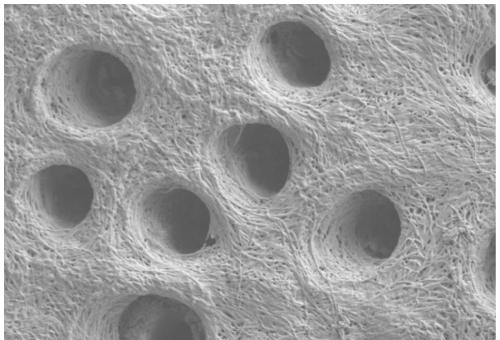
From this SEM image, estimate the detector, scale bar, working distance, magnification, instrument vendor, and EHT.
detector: SE2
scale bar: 1000 nm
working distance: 5.3 mm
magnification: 6 K X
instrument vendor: Zeiss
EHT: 3 kV